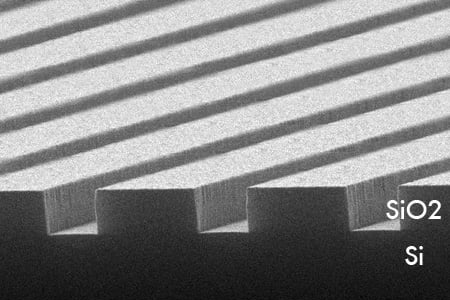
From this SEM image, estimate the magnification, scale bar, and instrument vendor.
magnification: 8 K X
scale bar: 2000 nm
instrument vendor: other